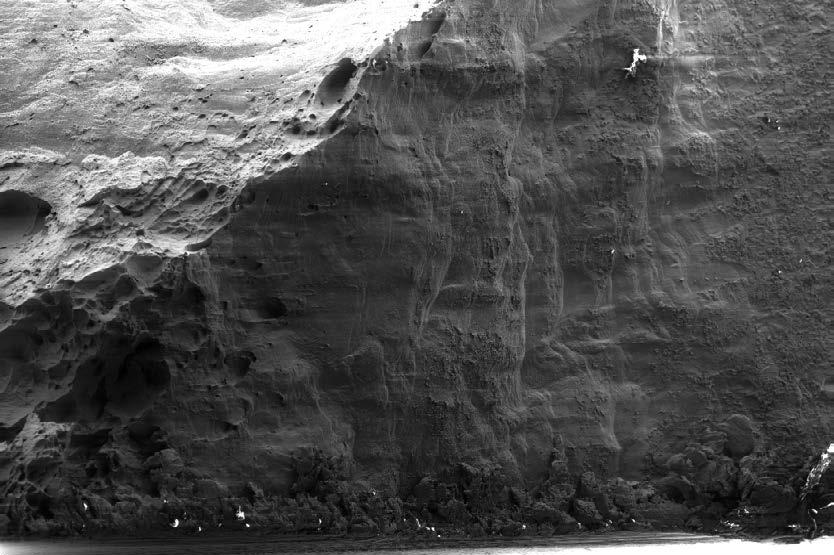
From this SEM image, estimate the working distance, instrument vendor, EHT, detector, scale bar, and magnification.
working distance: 8.7 mm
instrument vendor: Zeiss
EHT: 15 kV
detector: SE2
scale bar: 100000 nm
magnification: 0.1 K X